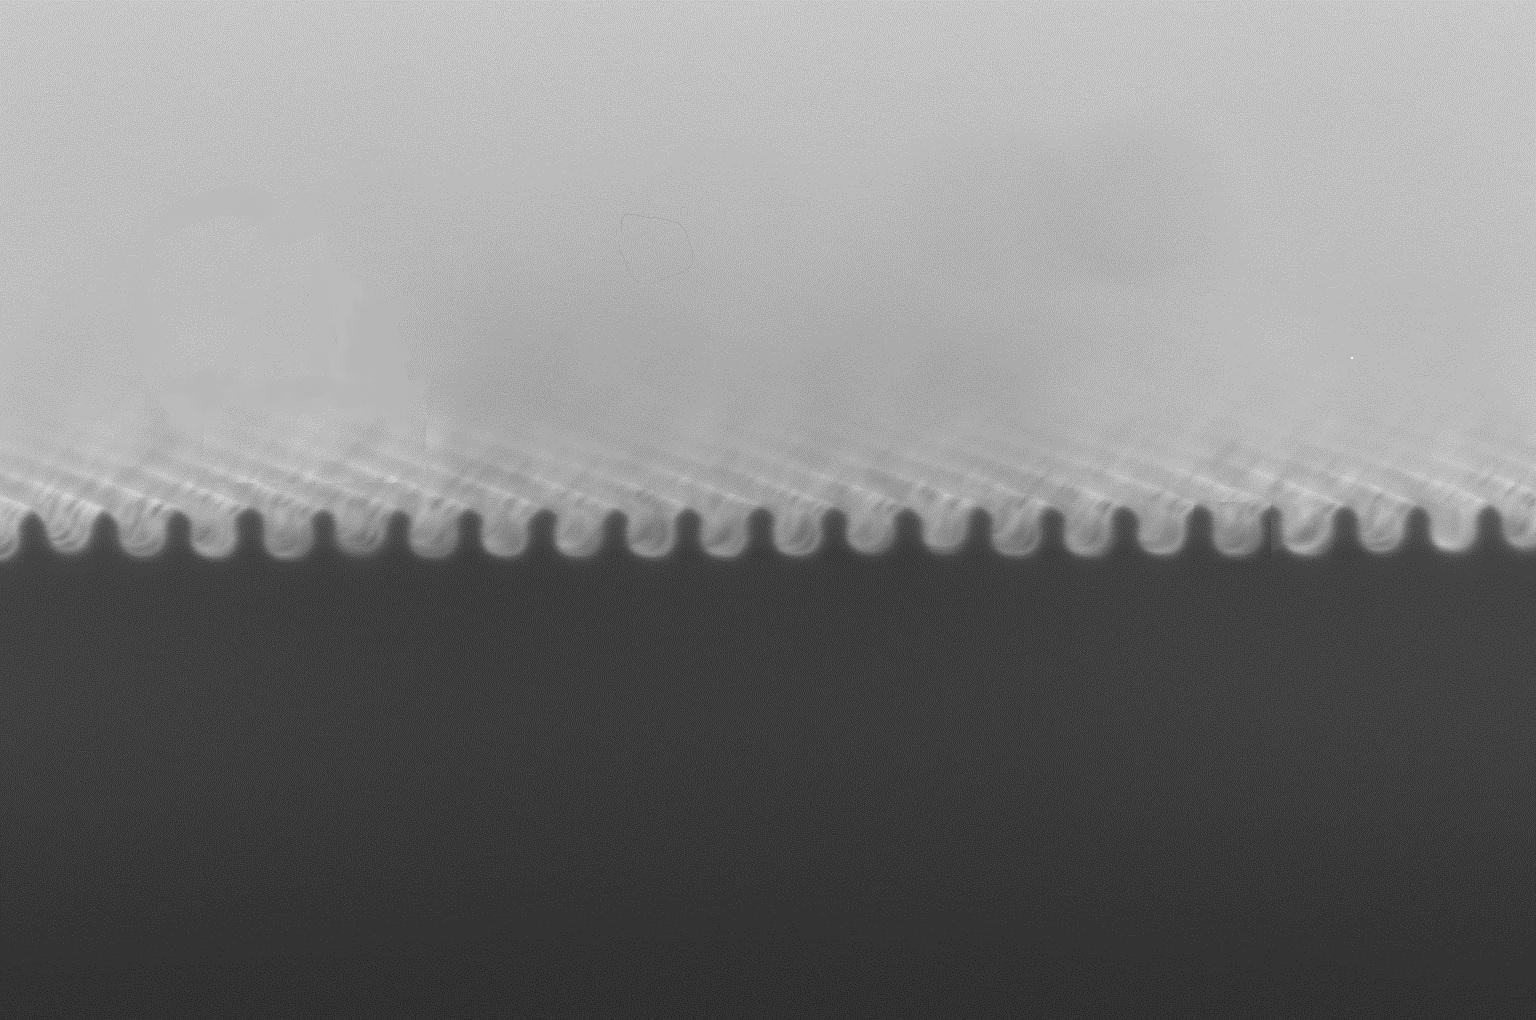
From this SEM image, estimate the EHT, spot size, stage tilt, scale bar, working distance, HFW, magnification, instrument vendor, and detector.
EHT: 20 kV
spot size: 4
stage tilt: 0°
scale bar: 1000 nm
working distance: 7.1 mm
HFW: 4.14 µm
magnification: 100 K X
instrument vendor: FEI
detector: ETD